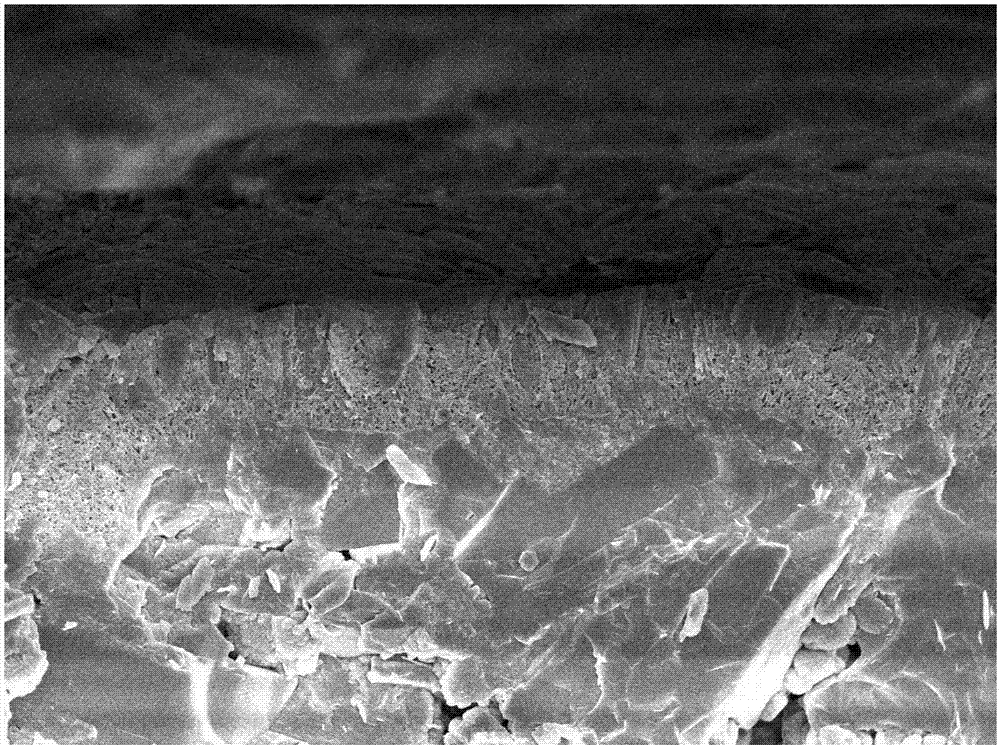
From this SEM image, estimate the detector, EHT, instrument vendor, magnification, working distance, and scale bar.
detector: SEI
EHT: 5 kV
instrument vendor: JEOL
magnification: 5 K X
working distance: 7.9 mm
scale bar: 1000 nm